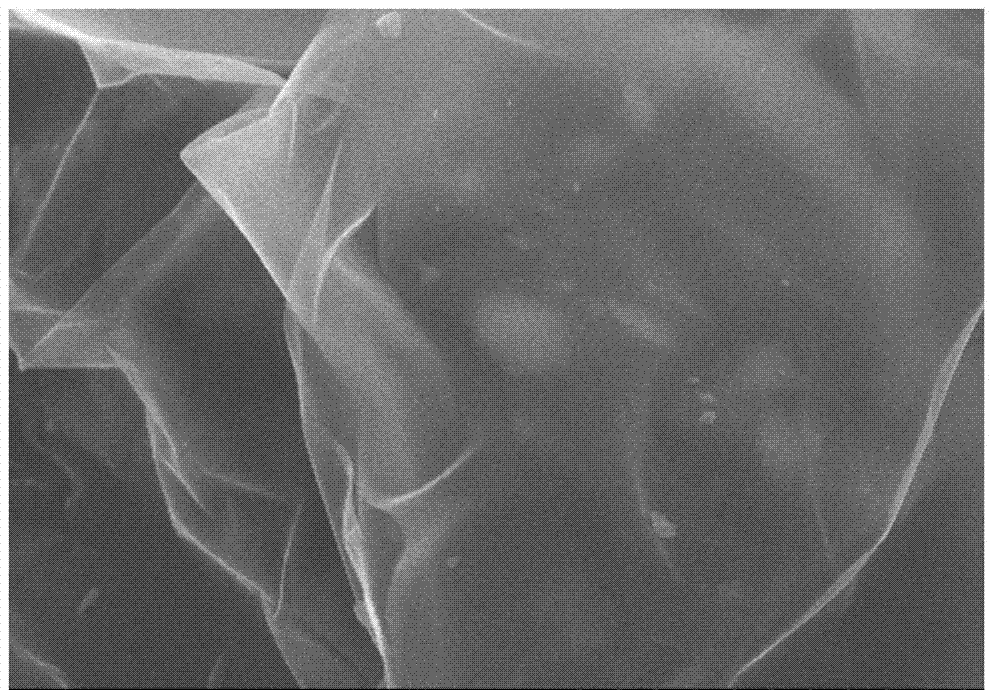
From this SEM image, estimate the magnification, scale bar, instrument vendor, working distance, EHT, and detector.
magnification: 10 K X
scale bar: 5000 nm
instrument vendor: Hitachi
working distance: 9.7 mm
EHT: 10 kV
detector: SE(M)